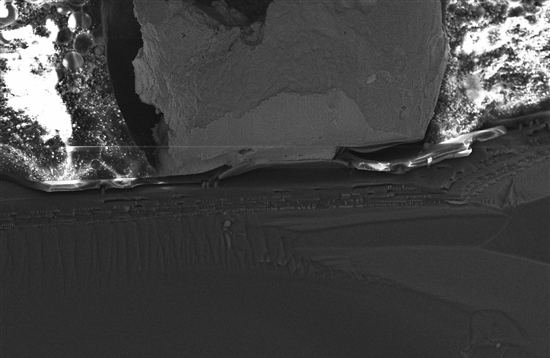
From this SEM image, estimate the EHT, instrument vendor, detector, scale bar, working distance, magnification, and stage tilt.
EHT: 15 kV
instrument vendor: Zeiss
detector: InLens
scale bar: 20000 nm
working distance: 4 mm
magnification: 1.21 K X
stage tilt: -15°